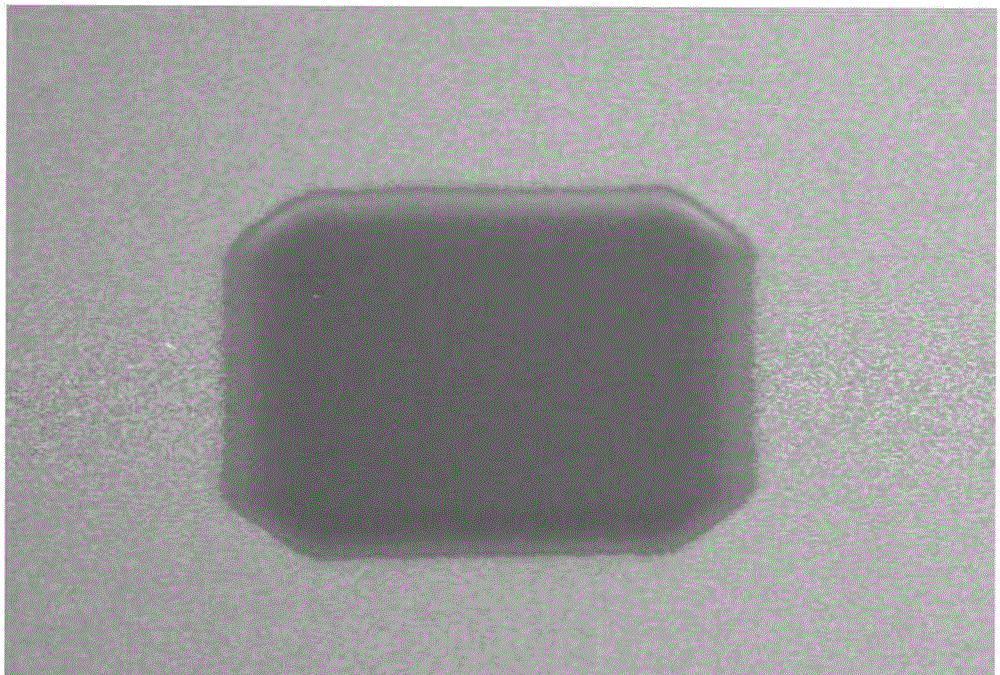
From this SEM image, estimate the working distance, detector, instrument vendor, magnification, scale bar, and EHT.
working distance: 11.9 mm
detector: SE(U)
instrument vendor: Hitachi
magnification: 1.1 K X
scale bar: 50000 nm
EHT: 15 kV